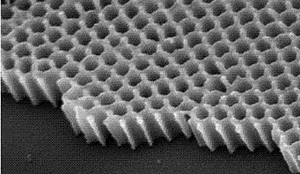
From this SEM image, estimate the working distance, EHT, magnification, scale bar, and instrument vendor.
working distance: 4.3 mm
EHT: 5 kV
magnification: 80 K X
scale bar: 200 nm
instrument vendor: FEI